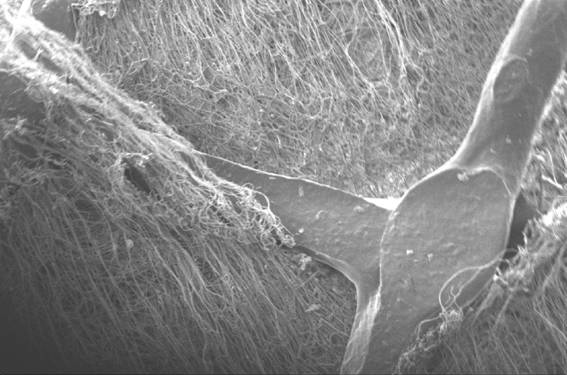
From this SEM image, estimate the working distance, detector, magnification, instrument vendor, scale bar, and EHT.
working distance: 8.5 mm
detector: SE1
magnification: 0.05 K X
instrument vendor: Zeiss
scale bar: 200000 nm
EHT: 10 kV